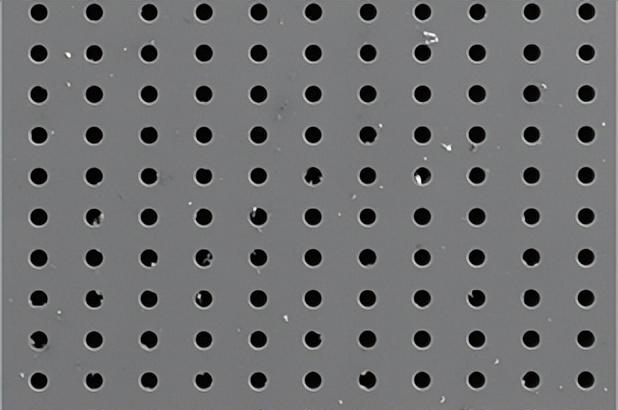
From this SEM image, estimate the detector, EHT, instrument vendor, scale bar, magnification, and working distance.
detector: SE2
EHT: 20 kV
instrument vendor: Zeiss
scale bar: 200000 nm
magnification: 0.05 K X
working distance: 16.9 mm